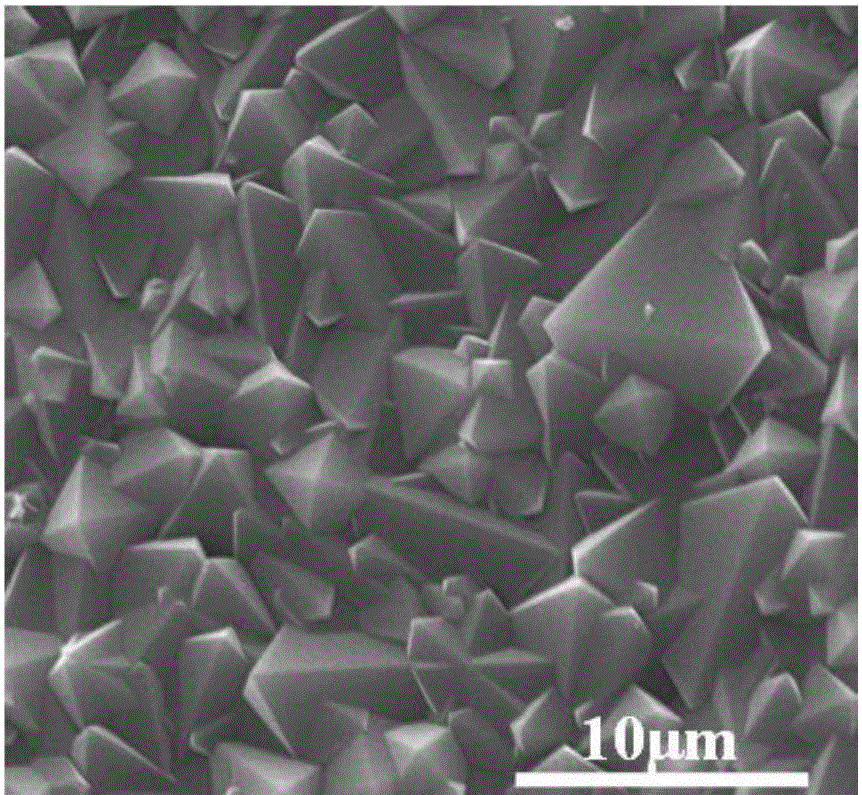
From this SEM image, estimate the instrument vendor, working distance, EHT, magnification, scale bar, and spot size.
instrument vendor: FEI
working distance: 15.3 mm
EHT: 20 kV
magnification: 10 K X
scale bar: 10000 nm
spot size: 3